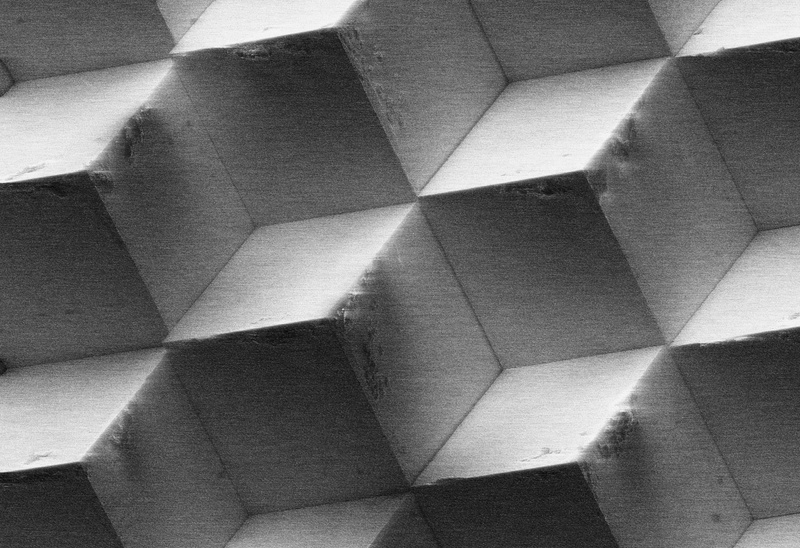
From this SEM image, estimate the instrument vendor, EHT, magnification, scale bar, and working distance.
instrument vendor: other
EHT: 8.5 kV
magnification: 0.115 K X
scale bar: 20000 nm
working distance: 35 mm